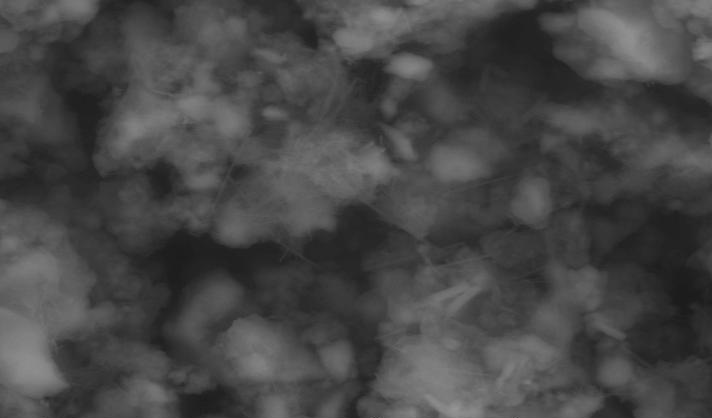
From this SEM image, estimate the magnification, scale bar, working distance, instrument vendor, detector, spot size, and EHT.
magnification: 1.234 K X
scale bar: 20000 nm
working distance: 14.1 mm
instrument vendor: FEI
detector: GSE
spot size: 5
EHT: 30 kV